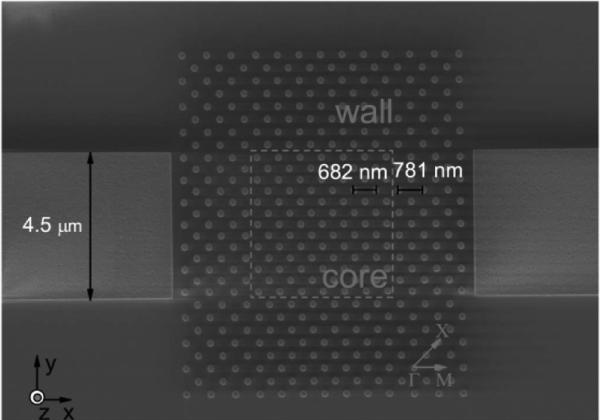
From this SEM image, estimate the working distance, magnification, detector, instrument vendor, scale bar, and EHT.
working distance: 6 mm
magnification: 15.92 K X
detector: SE2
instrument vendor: Zeiss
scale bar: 2000 nm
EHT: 1.5 kV